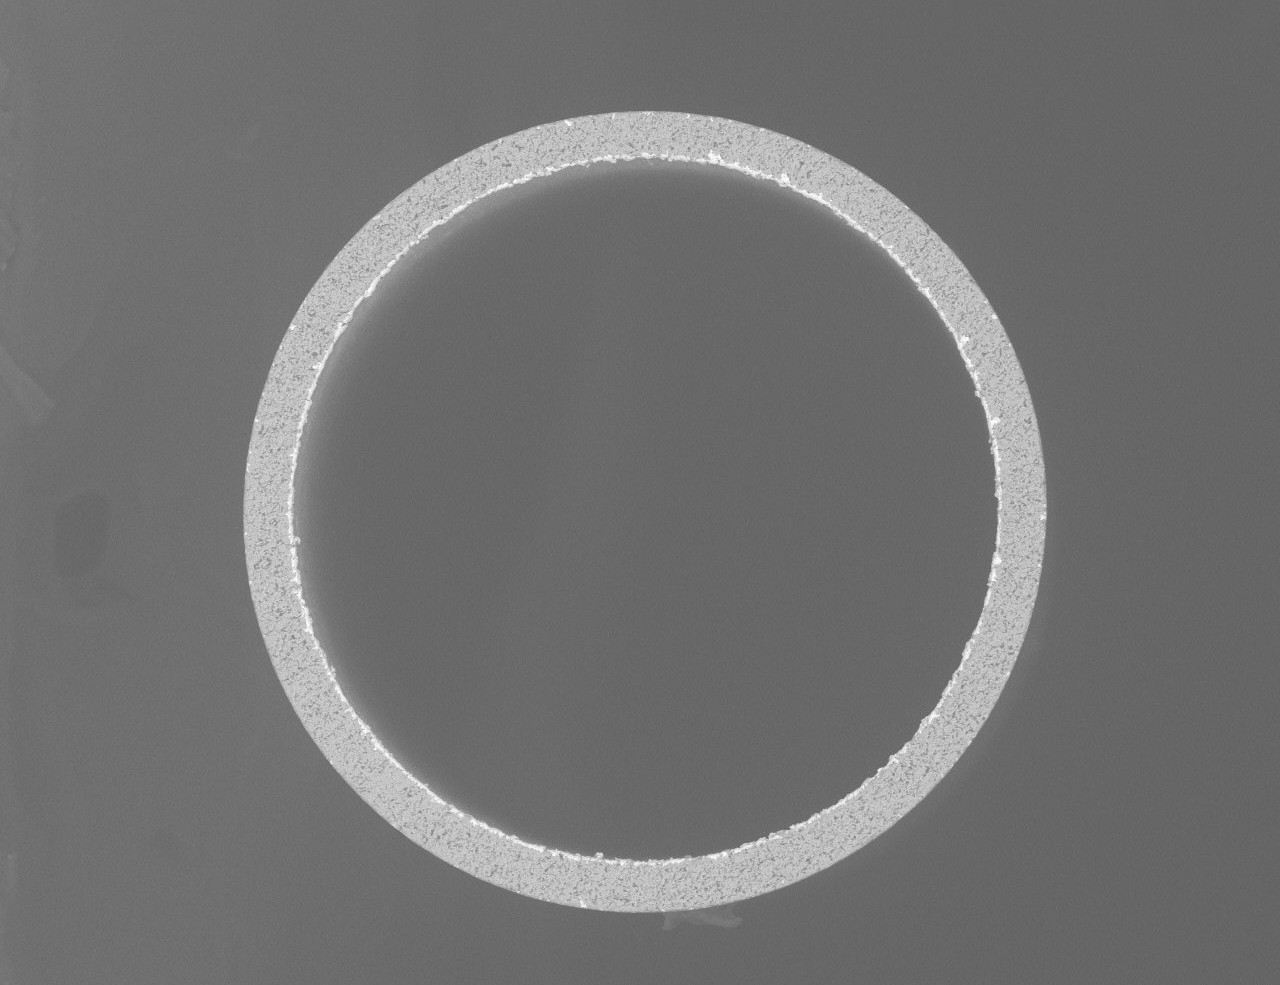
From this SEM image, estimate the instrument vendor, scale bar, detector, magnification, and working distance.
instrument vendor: Hitachi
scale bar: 200000 nm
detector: NL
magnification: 0.4 K X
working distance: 8.5 mm